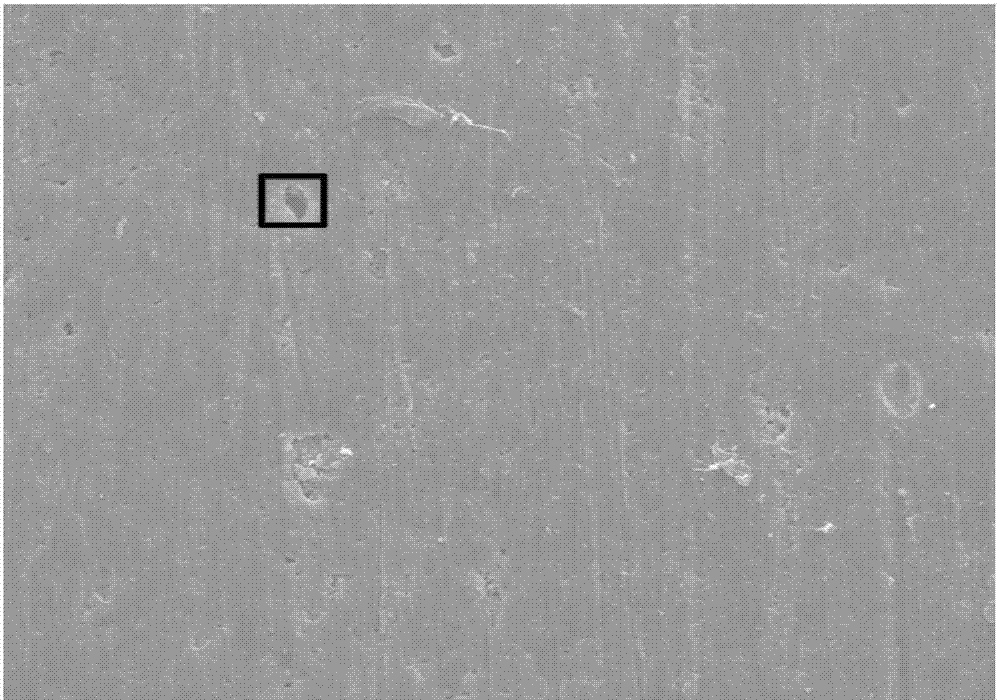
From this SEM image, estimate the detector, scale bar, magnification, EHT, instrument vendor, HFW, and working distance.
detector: ETD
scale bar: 50000 nm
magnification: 1 K X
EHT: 15 kV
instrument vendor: FEI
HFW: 127 µm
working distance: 10.6 mm